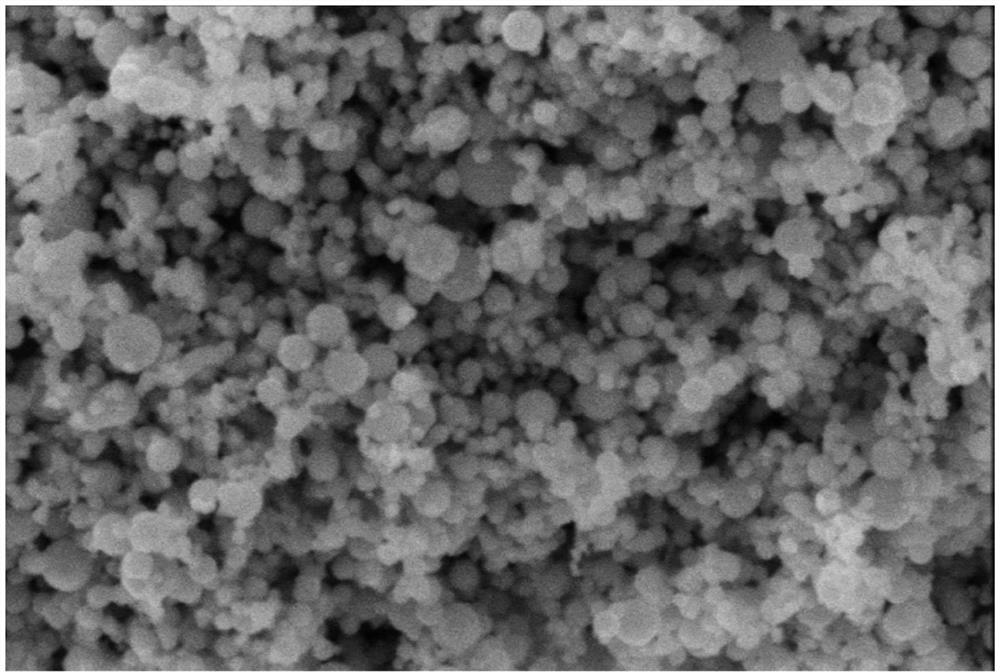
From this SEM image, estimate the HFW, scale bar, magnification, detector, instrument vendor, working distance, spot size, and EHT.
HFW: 5.18 µm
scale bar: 1000 nm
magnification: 80 K X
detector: ETD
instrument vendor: FEI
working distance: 10.8 mm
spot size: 3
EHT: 10 kV